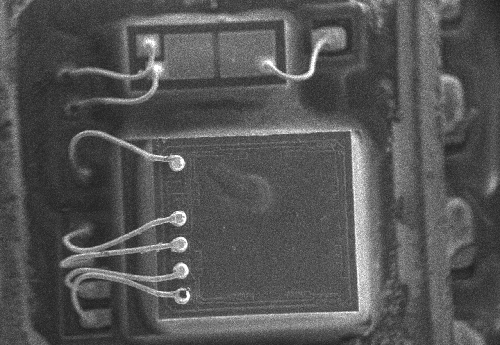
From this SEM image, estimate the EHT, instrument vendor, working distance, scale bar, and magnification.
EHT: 15 kV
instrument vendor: JEOL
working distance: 5.5 mm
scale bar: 200000 nm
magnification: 0.05 K X